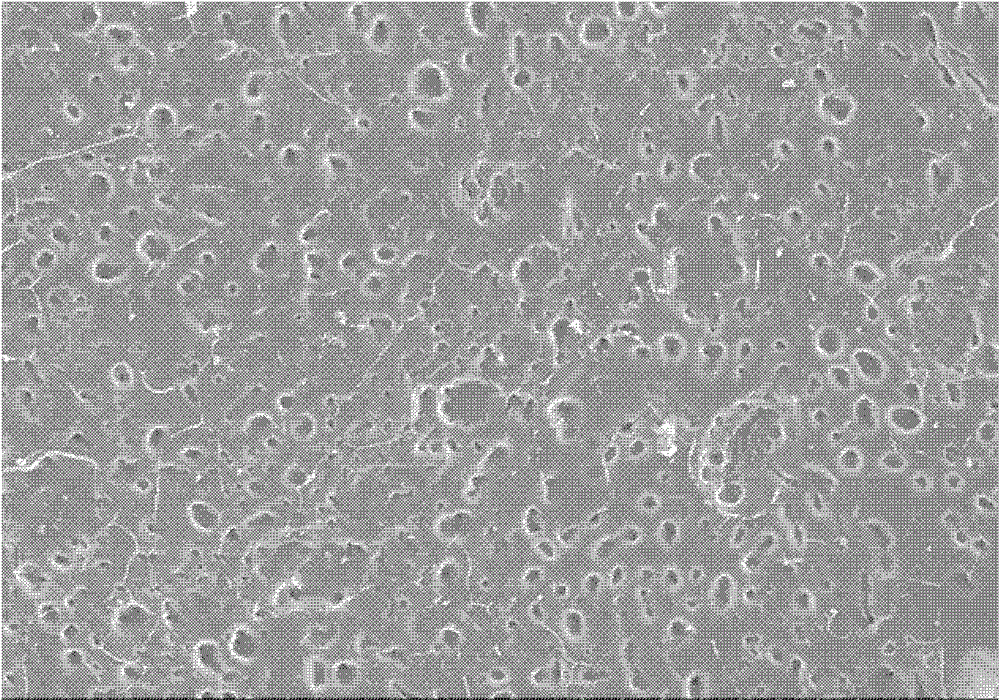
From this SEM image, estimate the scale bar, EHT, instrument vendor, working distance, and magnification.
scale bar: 500000 nm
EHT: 20 kV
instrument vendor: Hitachi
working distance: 14 mm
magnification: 0.1 K X